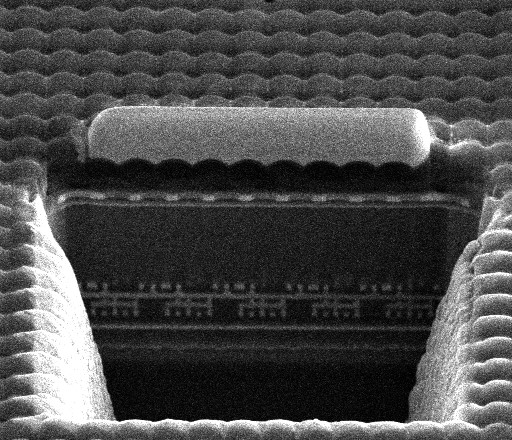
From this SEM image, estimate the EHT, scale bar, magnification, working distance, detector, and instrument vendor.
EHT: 10 kV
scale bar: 5000 nm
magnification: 8 K X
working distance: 4.2 mm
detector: ETD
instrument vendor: FEI